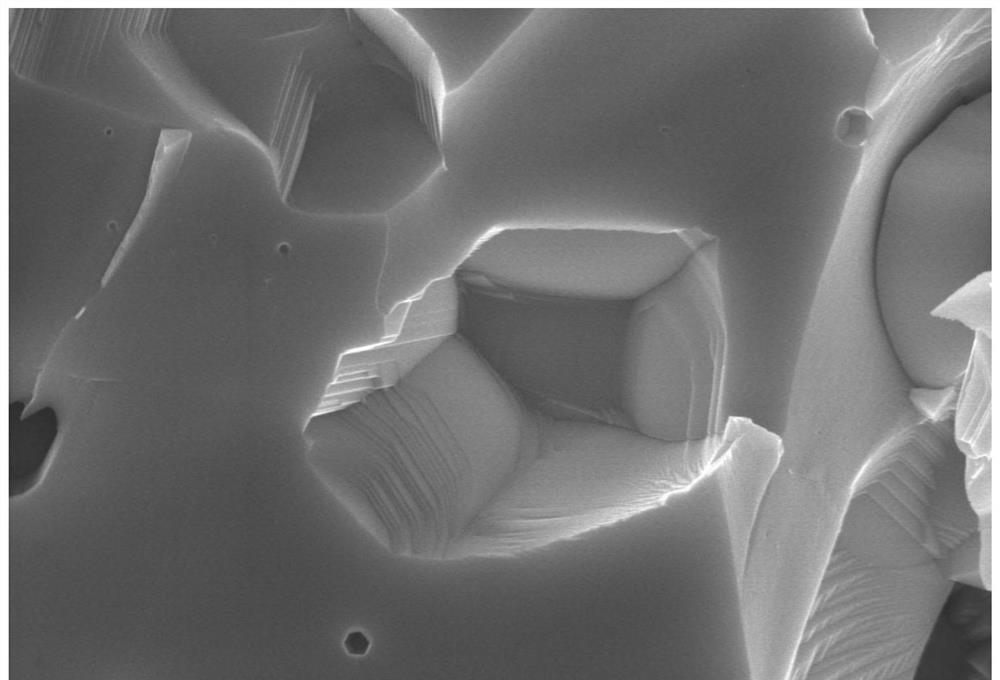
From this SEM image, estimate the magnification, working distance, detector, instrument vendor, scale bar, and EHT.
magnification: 20 K X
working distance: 15.1 mm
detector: SE(U)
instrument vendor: Hitachi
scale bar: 2000 nm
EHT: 15 kV